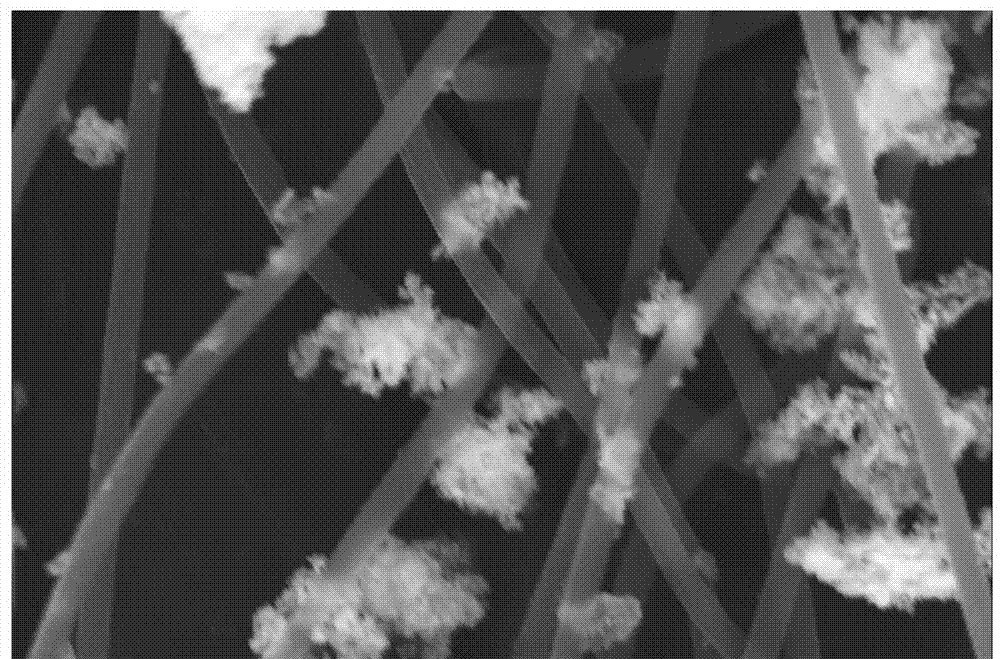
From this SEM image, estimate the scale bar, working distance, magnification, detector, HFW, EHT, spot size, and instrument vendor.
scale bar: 2000 nm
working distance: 9.7 mm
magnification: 20 K X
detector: SE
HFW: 10.4 µm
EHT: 20 kV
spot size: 5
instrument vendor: FEI